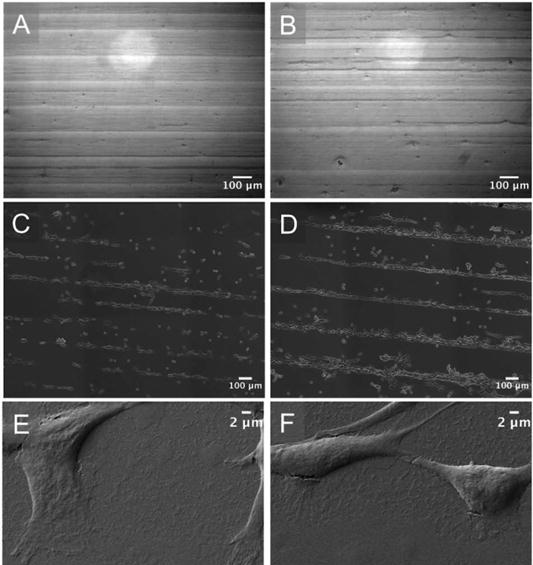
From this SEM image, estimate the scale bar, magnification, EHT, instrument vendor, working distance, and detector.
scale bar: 2000 nm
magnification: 2 K X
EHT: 2 kV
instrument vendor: Zeiss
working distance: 4.3 mm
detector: SE2